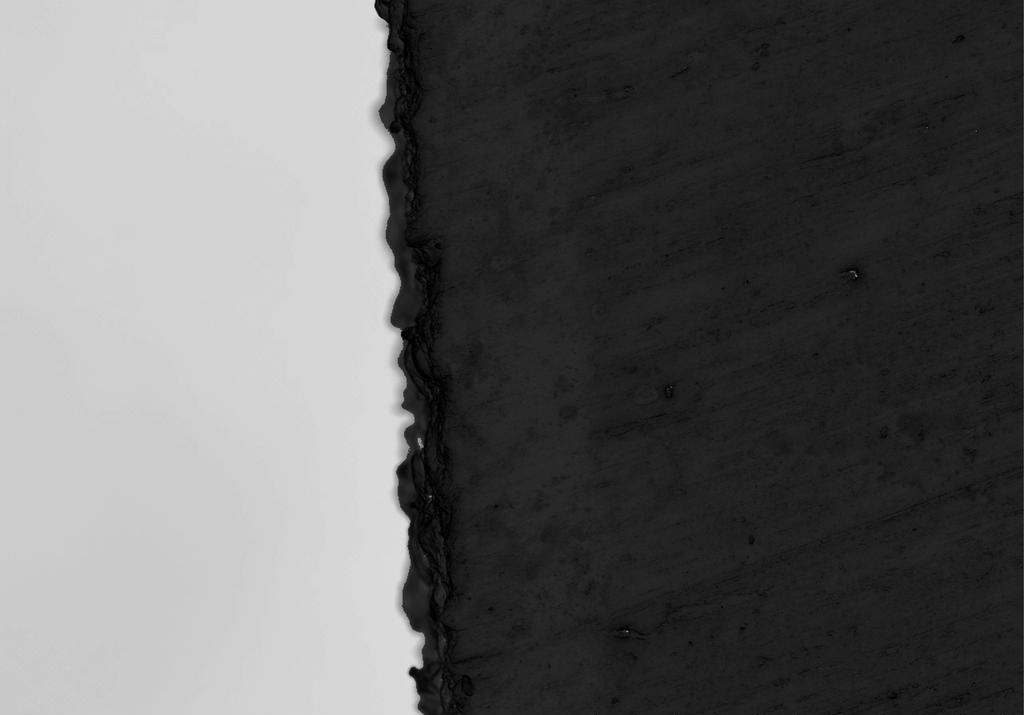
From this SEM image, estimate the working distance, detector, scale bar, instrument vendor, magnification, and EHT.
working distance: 14.4 mm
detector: SE(U)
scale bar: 50000 nm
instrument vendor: Hitachi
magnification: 1 K X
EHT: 15 kV